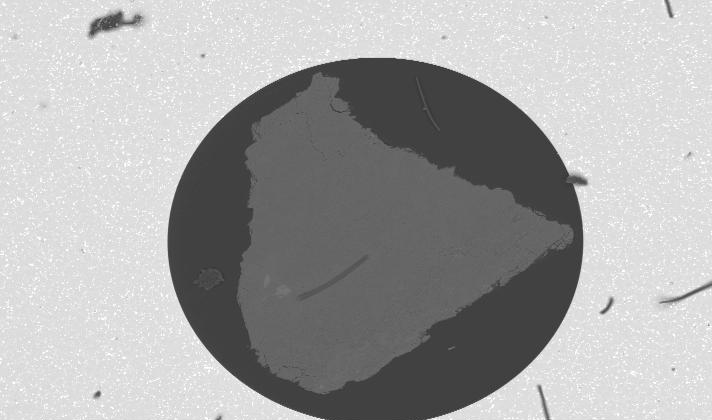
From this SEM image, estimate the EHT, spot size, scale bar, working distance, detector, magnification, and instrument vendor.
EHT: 25 kV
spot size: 4.2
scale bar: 500000 nm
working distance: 10 mm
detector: BSE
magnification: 0.05 K X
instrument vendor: FEI